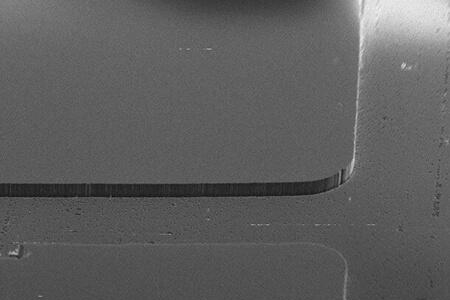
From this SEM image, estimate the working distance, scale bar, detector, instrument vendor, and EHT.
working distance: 2.6 mm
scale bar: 10000 nm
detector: SE2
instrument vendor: Zeiss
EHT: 15 kV